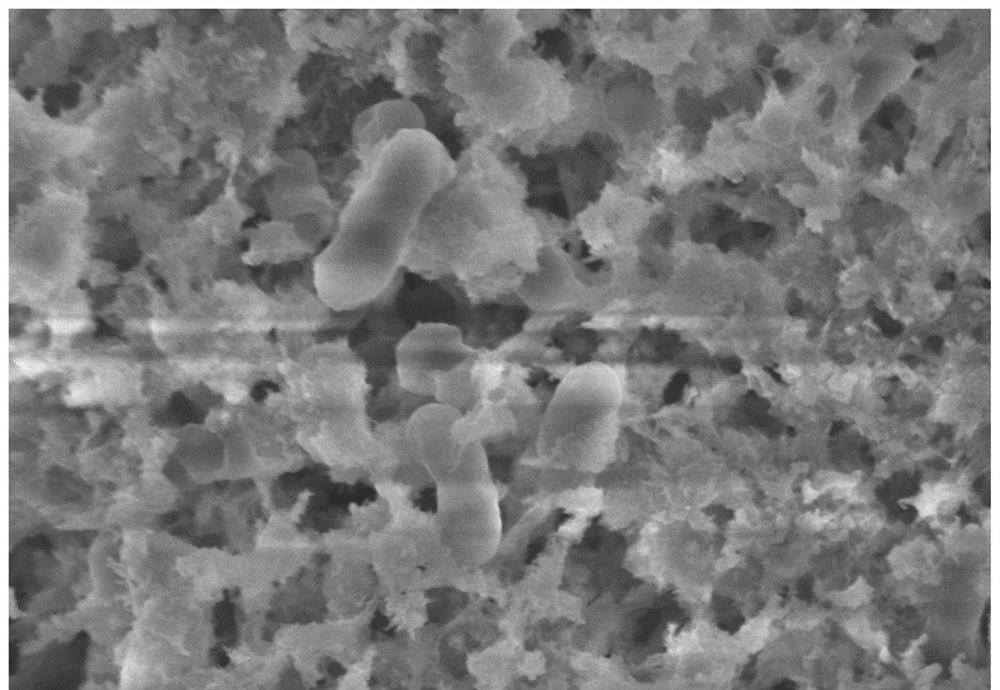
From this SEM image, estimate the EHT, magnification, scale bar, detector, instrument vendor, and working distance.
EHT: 5 kV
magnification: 10 K X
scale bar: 5000 nm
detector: SE(M)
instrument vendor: Hitachi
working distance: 7.6 mm